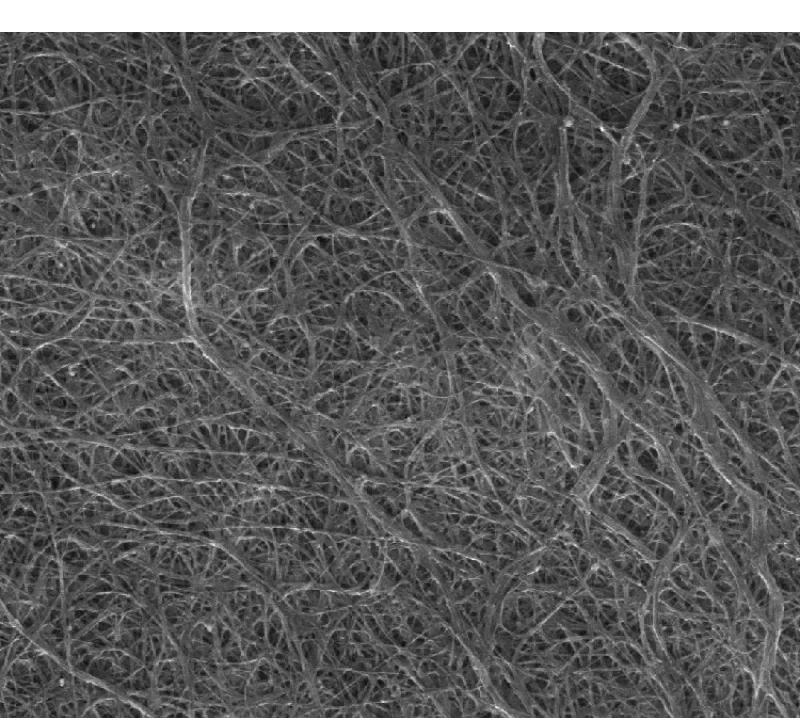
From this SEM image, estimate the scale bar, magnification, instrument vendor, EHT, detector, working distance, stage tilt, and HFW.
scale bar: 2000 nm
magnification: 30 K X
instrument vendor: FEI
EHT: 10 kV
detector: ETD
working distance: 11.7 mm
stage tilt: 0°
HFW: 9.95 µm